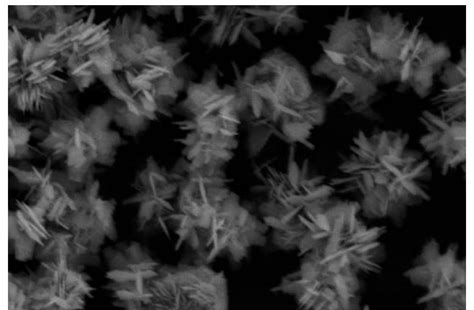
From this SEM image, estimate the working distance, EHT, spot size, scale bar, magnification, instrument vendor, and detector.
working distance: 7.1 mm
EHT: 13 kV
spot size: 4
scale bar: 5000 nm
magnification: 10 K X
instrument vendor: other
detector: SE